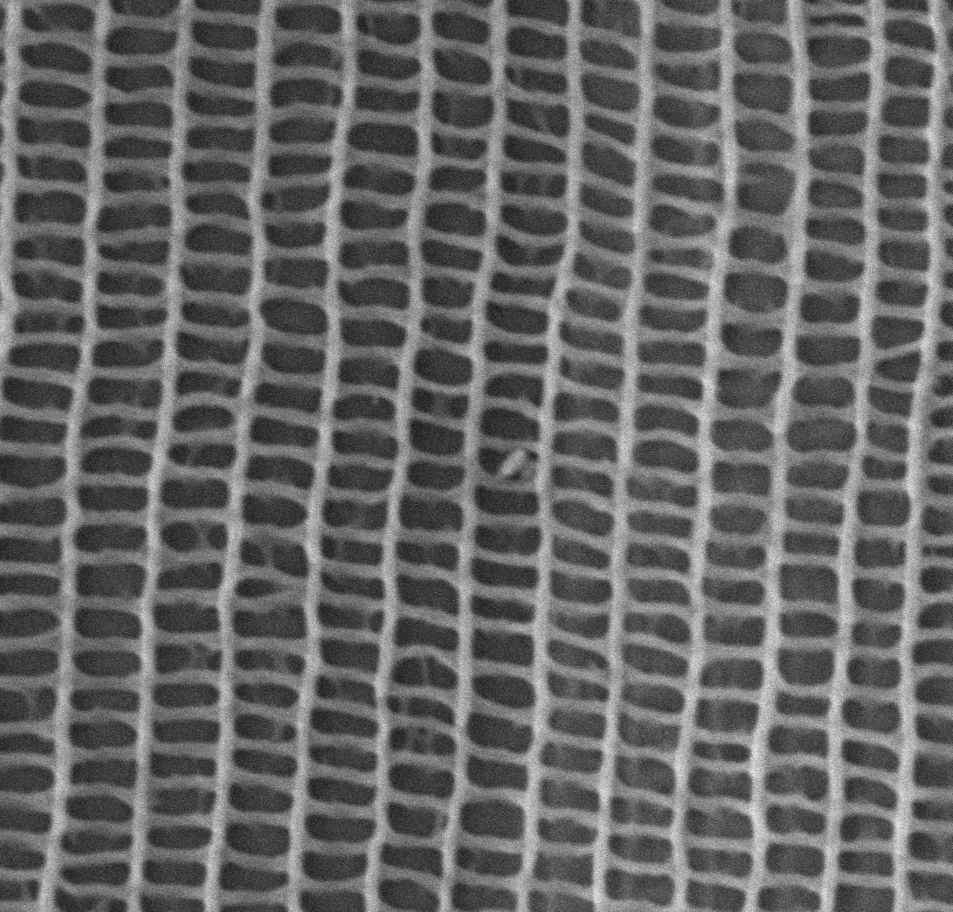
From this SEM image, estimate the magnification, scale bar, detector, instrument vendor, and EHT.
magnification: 20 K X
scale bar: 2000 nm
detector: SE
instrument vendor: other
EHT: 15 kV